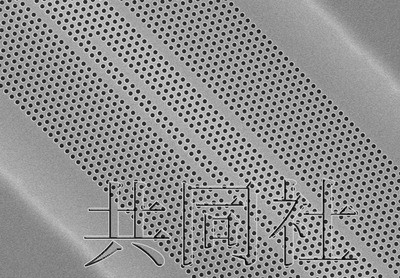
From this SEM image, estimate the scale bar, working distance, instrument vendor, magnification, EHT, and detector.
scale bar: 5000 nm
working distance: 8.1 mm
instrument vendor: Hitachi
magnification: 6 K X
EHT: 10 kV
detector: SE(M)